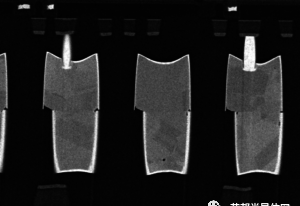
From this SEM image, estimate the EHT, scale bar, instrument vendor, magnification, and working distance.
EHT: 3 kV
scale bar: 2000 nm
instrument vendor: Hitachi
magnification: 20 K X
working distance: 5 mm